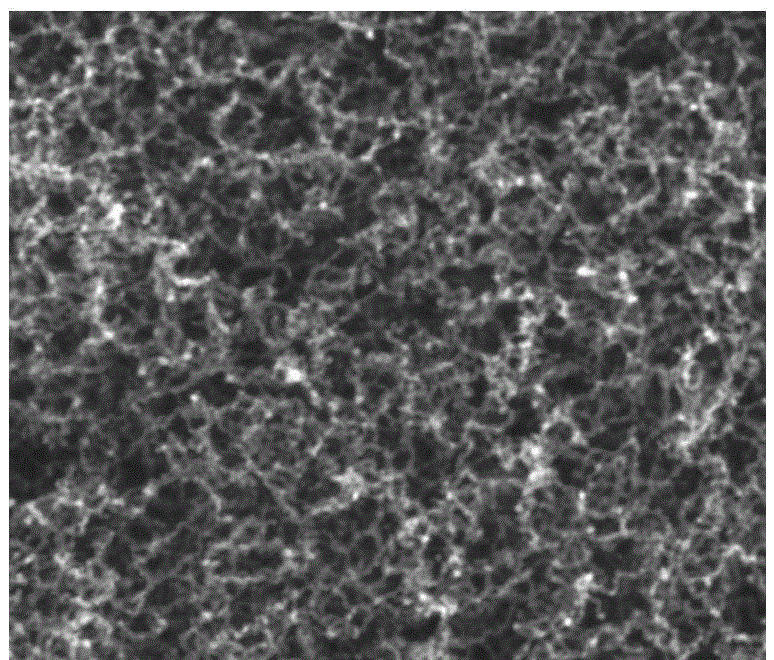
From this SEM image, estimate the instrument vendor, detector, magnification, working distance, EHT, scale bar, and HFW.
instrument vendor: FEI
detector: TLD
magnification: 100 K X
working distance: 5.3 mm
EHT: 15 kV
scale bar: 400 nm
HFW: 1.28 µm